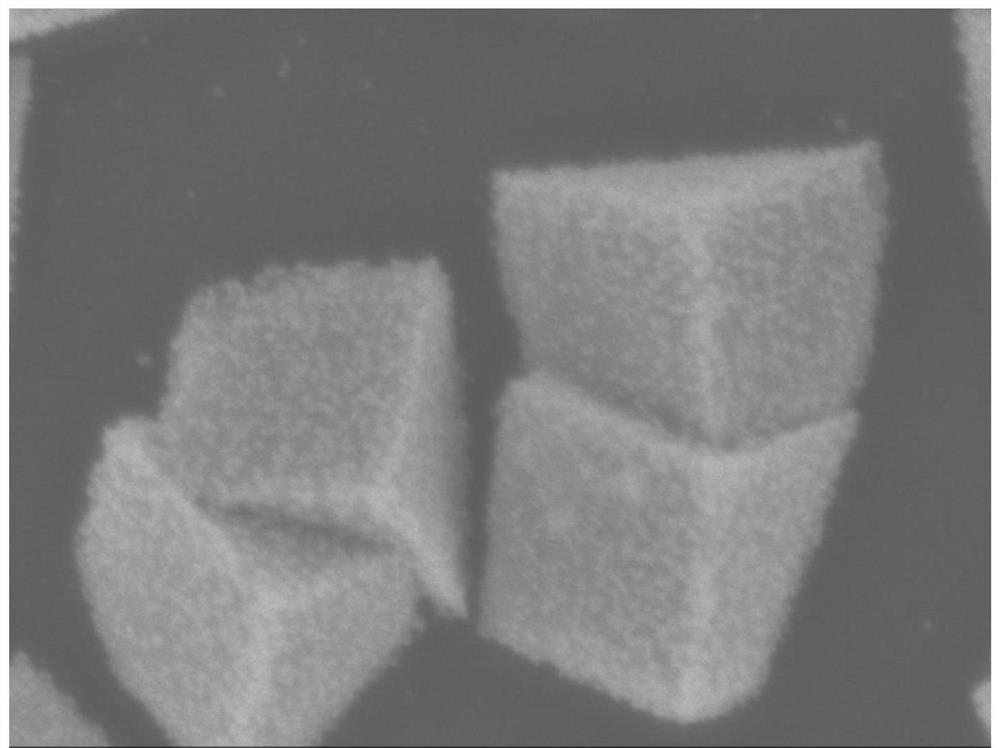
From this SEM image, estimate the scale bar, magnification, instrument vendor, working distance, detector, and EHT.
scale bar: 100 nm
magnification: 45 K X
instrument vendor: JEOL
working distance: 7.2 mm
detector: SEI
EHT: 5 kV